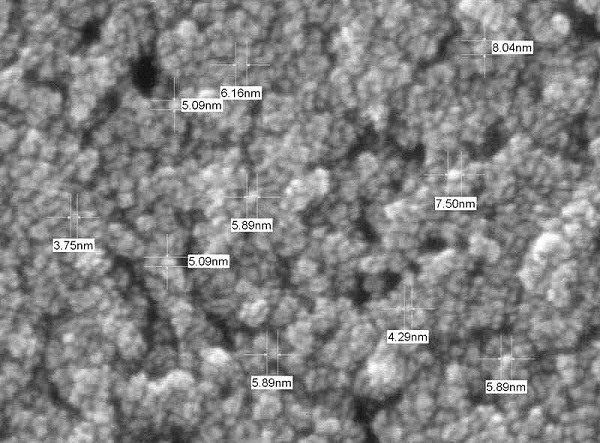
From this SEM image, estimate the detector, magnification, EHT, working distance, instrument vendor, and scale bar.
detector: SEI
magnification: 350 K X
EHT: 10 kV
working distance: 4.5 mm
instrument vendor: JEOL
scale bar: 10 nm